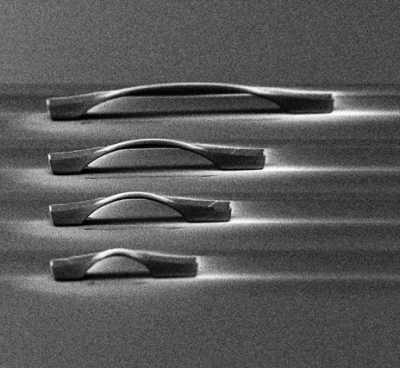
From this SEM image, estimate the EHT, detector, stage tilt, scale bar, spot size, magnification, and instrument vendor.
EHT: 30 kV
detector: ETD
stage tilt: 5°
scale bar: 20000 nm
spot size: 5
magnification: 5 K X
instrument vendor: FEI